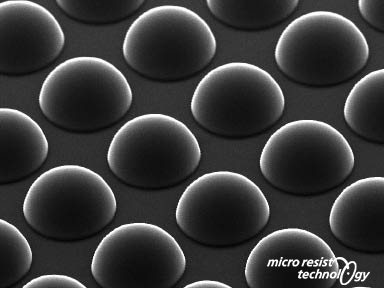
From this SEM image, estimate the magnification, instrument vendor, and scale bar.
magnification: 0.5 K X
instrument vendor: other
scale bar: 60000 nm